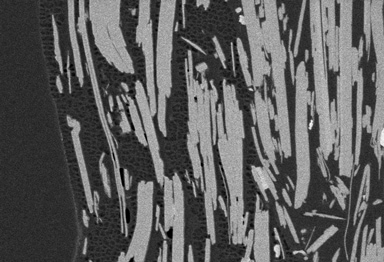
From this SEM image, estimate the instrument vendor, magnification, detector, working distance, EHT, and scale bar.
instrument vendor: Hitachi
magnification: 30 K X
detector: LA100(U)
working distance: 2.5 mm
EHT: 0.8 kV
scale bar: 1000 nm